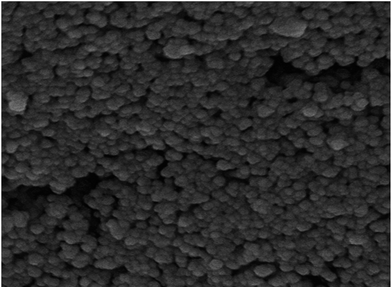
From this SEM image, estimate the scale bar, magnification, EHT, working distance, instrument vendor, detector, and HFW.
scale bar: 200 nm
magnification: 100 K X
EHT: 10 kV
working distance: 1.35 mm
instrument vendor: Tescan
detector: InBeam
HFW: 1.27 µm